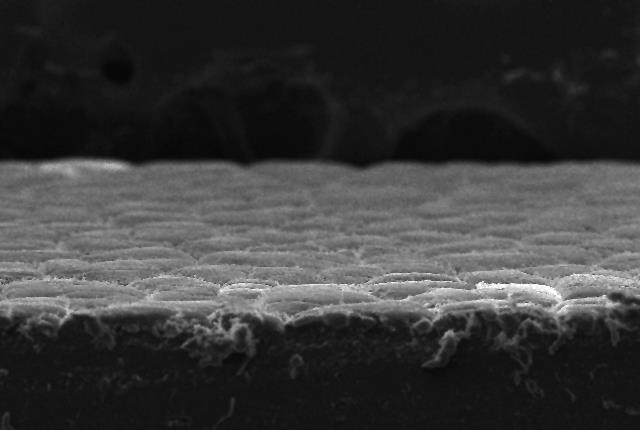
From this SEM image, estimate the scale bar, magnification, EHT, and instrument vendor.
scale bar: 100000 nm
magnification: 0.15 K X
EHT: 20 kV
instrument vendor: JEOL